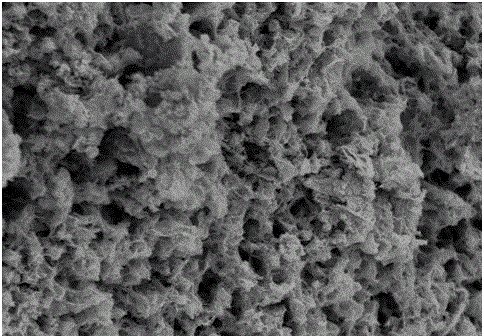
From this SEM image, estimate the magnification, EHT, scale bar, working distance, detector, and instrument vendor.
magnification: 10 K X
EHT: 5 kV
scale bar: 5000 nm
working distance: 9 mm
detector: SE(M)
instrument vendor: Hitachi